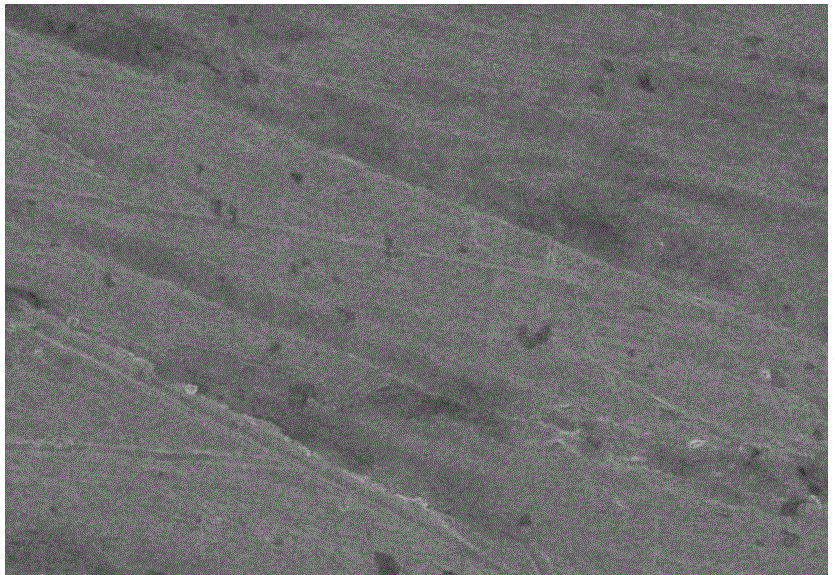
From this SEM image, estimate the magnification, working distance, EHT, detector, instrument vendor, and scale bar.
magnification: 10 K X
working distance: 11.2 mm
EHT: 3 kV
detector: SE(U)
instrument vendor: Hitachi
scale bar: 5000 nm